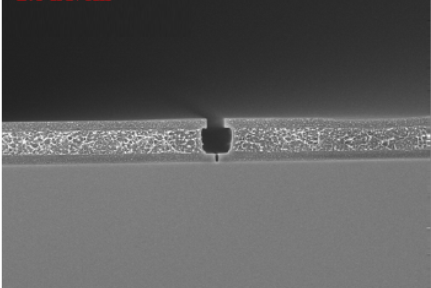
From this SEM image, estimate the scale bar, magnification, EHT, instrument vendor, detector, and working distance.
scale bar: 1000 nm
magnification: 10 K X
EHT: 3 kV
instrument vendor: JEOL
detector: SEI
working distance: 1.9 mm